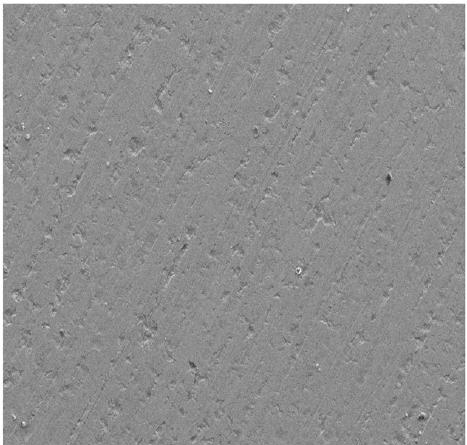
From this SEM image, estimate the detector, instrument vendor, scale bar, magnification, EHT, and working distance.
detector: SE2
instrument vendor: Zeiss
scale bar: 20000 nm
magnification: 0.5 K X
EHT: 15 kV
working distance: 7.9 mm